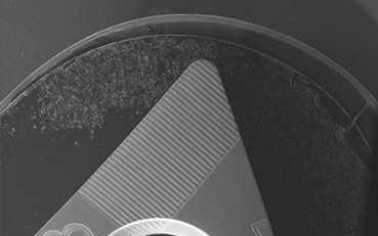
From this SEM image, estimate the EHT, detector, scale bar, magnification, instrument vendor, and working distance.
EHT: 20 kV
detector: SE1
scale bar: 1000000 nm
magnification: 0.013 K X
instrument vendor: Zeiss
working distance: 21 mm